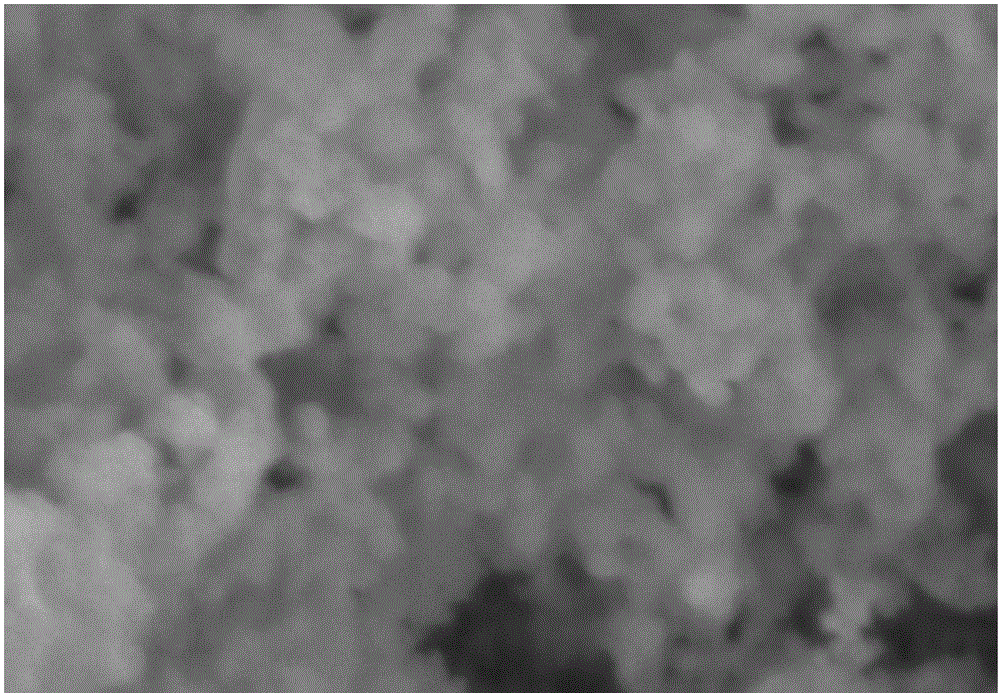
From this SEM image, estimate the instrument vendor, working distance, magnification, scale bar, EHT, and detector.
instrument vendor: Hitachi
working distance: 11.7 mm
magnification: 9.5 K X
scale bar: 5000 nm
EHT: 15 kV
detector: SE